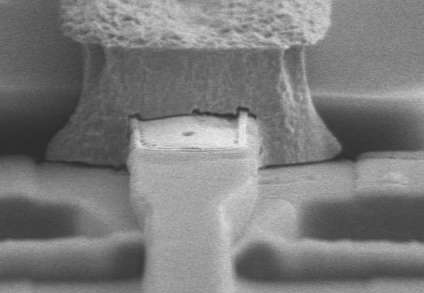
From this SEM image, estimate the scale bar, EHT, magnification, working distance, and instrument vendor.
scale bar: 5000 nm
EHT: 10 kV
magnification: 7 K X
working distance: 11.2 mm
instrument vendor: Hitachi